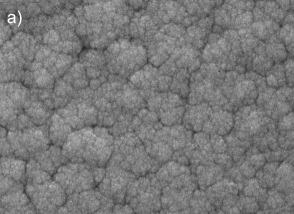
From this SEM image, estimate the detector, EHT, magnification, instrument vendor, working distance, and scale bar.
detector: SEI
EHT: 15 kV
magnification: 70 K X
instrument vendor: JEOL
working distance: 5.9 mm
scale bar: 100 nm